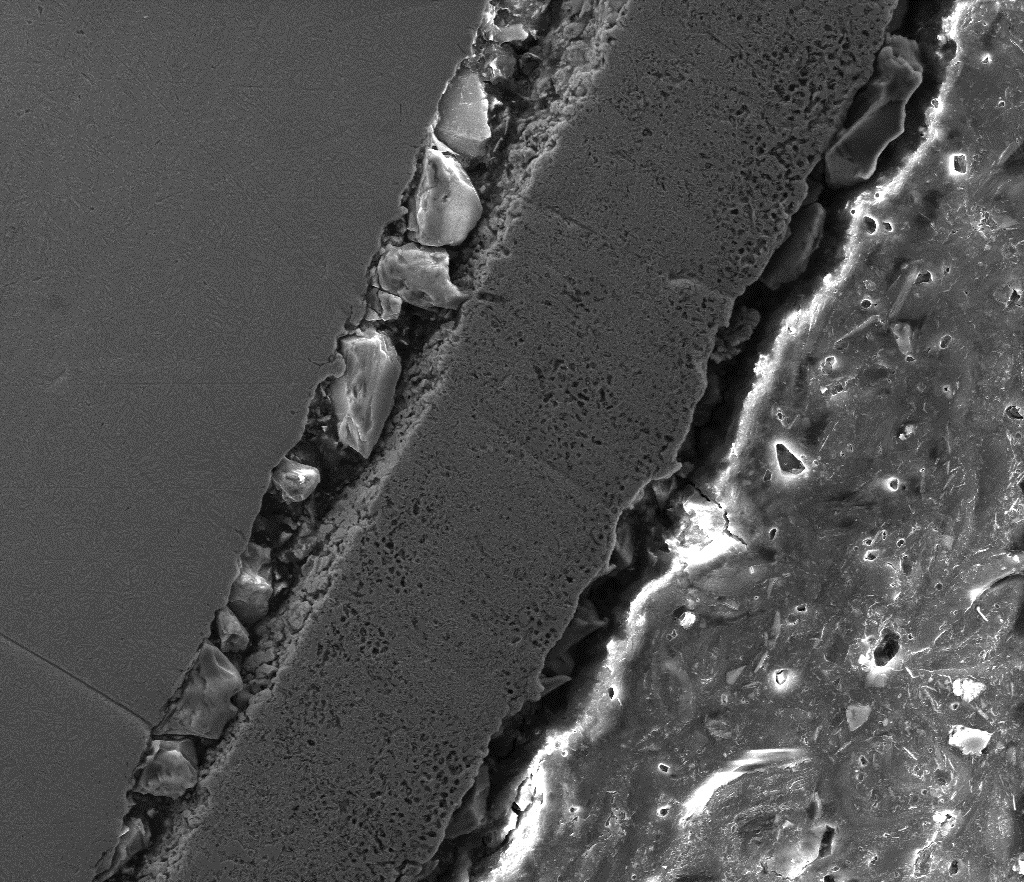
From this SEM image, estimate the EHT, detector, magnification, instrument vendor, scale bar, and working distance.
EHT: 20 kV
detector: ETD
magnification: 1 K X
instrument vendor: FEI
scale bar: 100000 nm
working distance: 9.6 mm